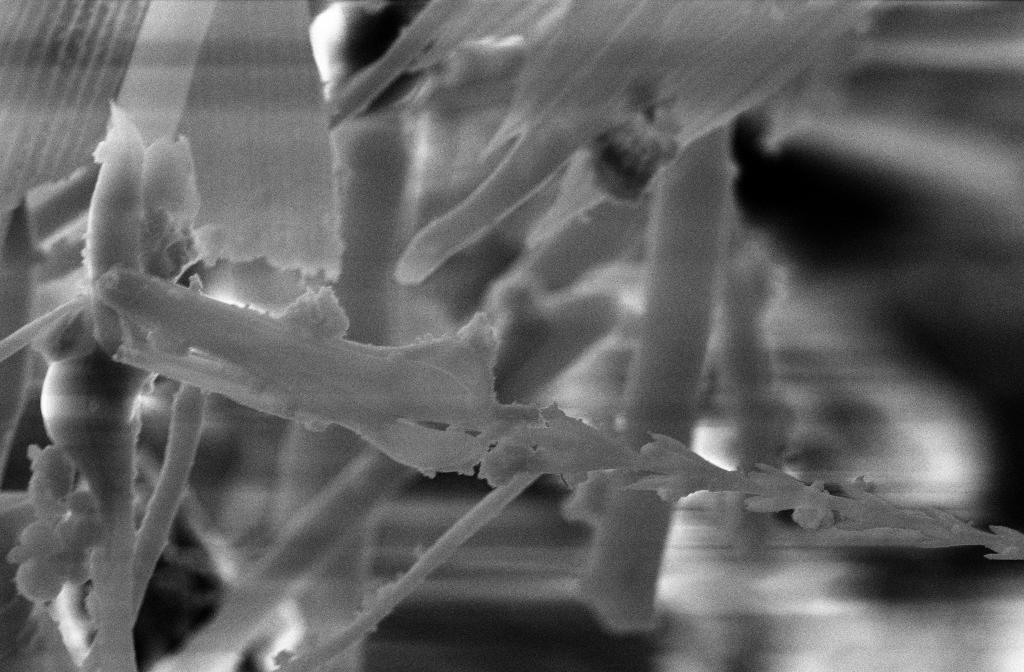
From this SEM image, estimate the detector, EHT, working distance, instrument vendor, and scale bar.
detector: SE2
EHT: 20 kV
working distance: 15 mm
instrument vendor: Zeiss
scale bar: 2000 nm